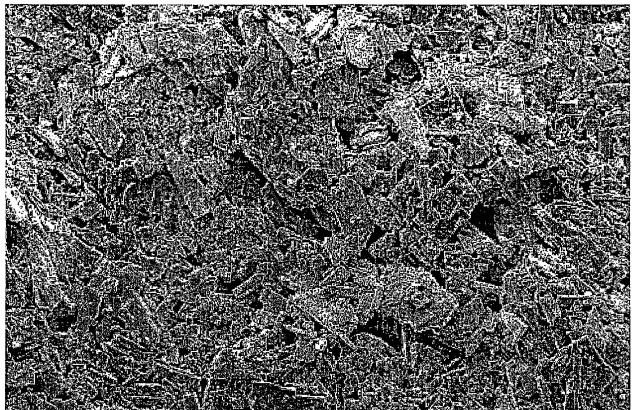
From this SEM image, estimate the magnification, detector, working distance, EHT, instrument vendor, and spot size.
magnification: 1 K X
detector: SE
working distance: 10 mm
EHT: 3 kV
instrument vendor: FEI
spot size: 2